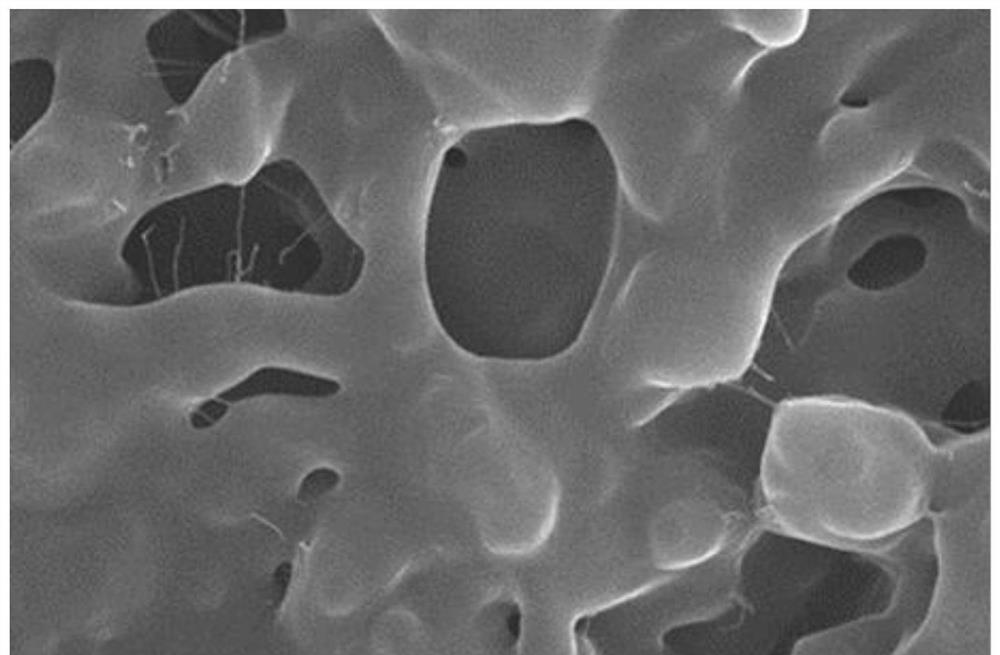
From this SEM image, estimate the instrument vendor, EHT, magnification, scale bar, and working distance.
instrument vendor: Hitachi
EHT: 30 kV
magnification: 5 K X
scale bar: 10000 nm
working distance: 7.7 mm